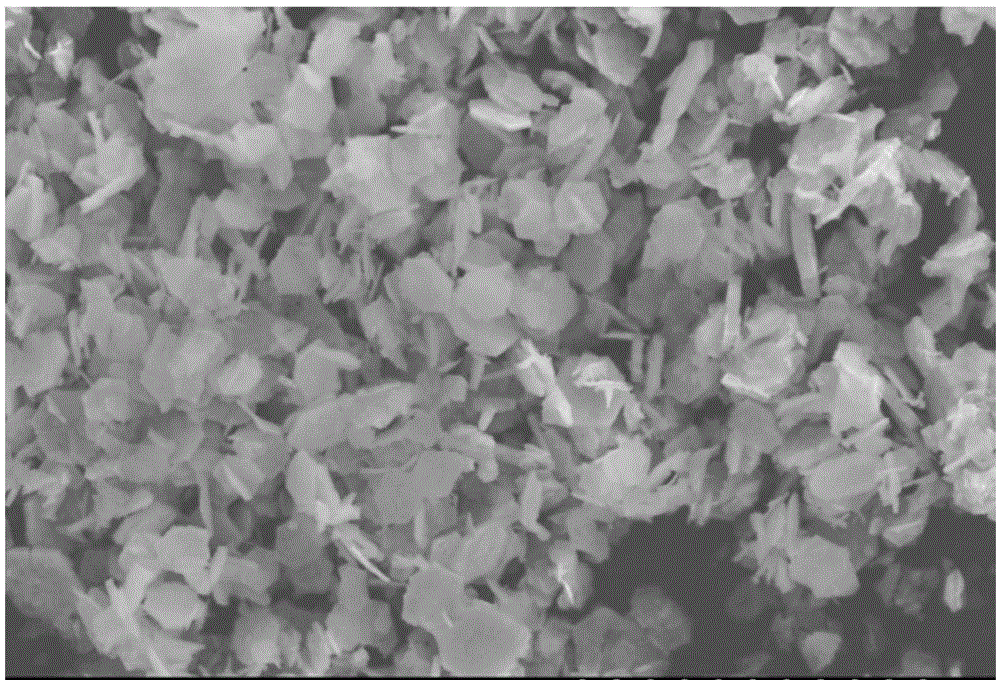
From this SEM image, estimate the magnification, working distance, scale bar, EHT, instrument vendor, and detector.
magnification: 1.5 K X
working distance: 5 mm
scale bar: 30000 nm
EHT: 20 kV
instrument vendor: Hitachi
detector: SE